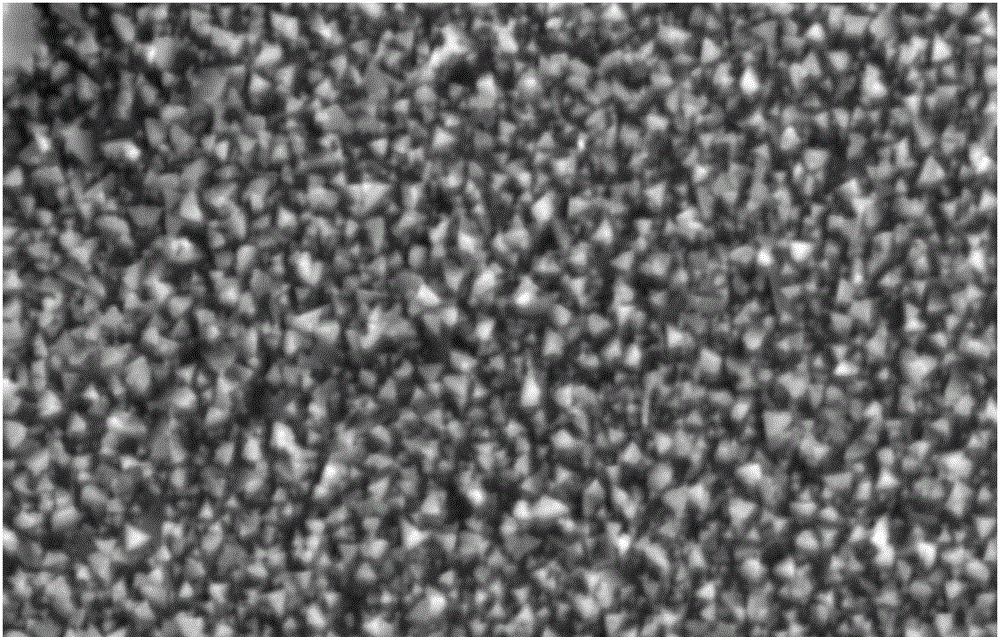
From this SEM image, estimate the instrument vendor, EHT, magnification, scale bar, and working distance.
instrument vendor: Zeiss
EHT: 15 kV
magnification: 15 K X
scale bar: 2000 nm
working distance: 11 mm